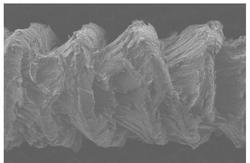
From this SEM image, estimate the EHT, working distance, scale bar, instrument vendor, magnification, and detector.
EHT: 3 kV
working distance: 11.2 mm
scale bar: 1000000 nm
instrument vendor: Hitachi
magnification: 0.03 K X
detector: SE(M)